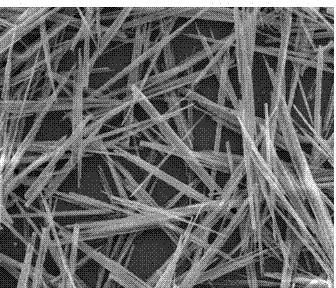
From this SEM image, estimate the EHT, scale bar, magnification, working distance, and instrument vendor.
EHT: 10 kV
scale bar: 20000 nm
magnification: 5 K X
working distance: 16.8 mm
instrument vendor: FEI